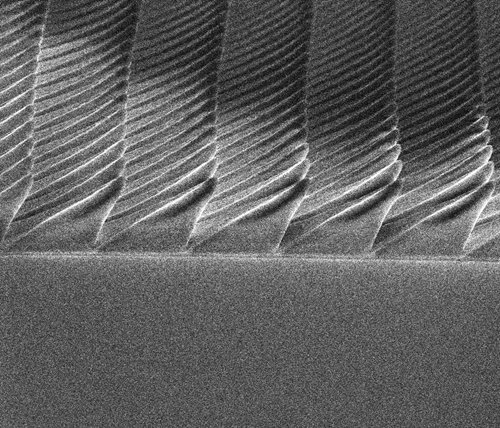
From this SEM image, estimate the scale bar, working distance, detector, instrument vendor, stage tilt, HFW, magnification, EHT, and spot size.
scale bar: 5000 nm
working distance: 4.8 mm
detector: ETD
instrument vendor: FEI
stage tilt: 45°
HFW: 27.1 µm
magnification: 11 K X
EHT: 5 kV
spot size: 2